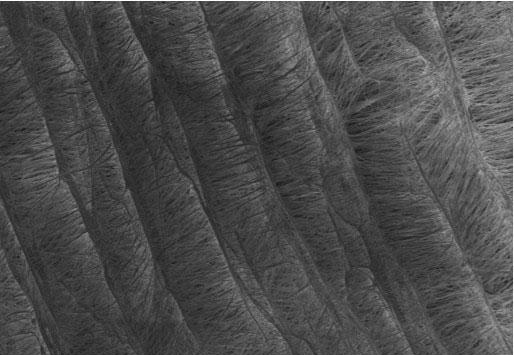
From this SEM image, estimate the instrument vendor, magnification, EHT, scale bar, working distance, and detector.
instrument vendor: Hitachi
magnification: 0.5 K X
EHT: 3 kV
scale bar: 100000 nm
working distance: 8.9 mm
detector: SE(M)